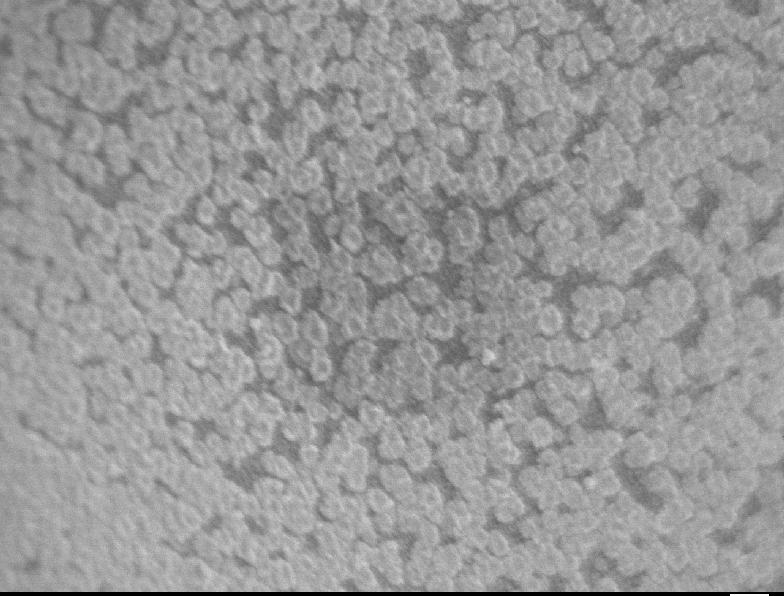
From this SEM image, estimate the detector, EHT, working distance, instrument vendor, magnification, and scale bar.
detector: SEI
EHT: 1 kV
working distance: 2 mm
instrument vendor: JEOL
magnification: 60 K X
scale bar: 100 nm